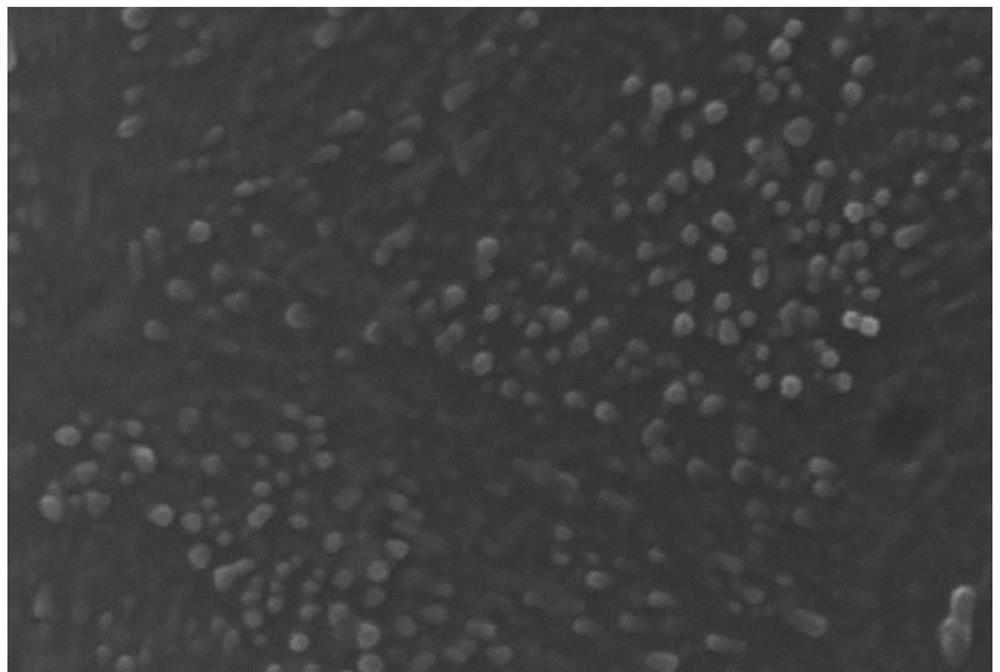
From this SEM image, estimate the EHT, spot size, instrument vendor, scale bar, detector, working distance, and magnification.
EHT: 15 kV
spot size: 5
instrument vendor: other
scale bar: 1000 nm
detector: SE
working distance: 5.9 mm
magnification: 50 K X